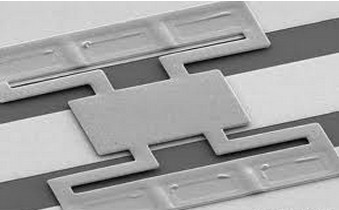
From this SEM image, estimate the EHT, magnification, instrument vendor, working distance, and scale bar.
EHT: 10 kV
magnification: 0.3 K X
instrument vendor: Hitachi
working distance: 38.4 mm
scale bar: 100000 nm